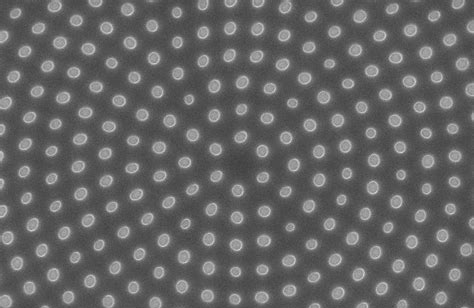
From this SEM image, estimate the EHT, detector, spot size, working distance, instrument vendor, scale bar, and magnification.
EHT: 5 kV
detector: TLD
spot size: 3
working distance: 5.2 mm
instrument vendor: FEI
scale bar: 3000 nm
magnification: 24 K X